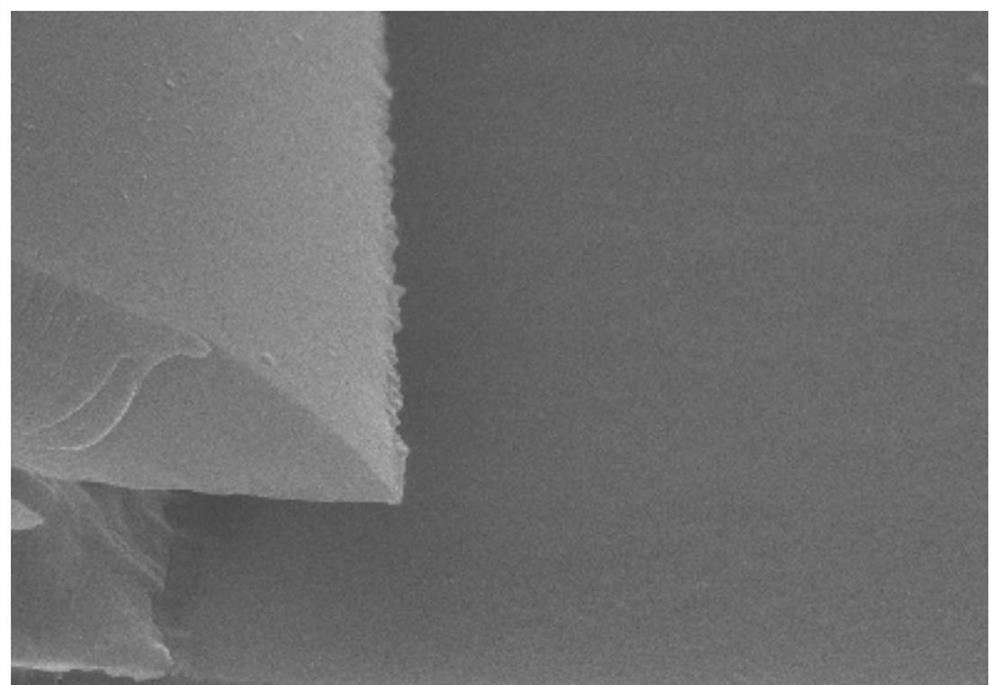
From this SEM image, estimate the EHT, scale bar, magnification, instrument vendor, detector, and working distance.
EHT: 10 kV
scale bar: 1000 nm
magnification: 30 K X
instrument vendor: Hitachi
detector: SE(U)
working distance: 13.9 mm